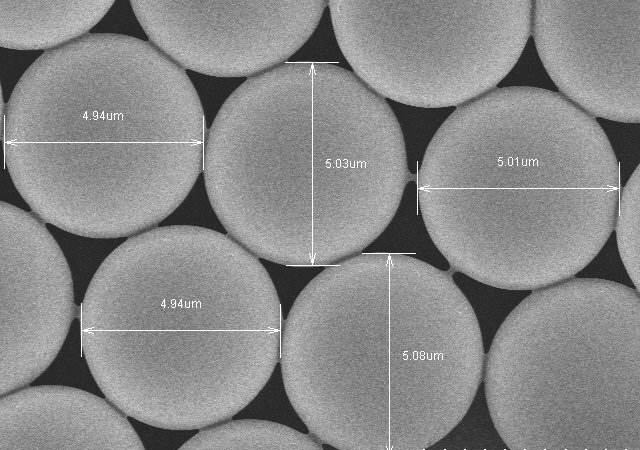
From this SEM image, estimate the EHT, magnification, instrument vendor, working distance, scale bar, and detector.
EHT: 4 kV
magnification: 8 K X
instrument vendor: Hitachi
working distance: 8.5 mm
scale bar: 5000 nm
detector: SE(M)